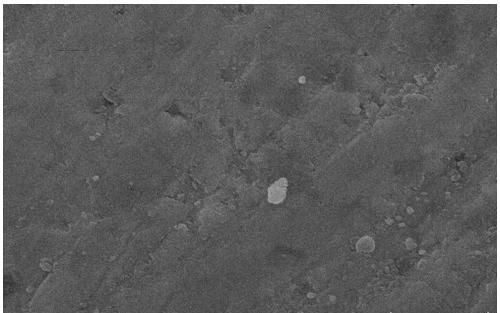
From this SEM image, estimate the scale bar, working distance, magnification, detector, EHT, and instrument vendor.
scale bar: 10000 nm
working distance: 8.5 mm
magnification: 5 K X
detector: SE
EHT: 30 kV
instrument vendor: other